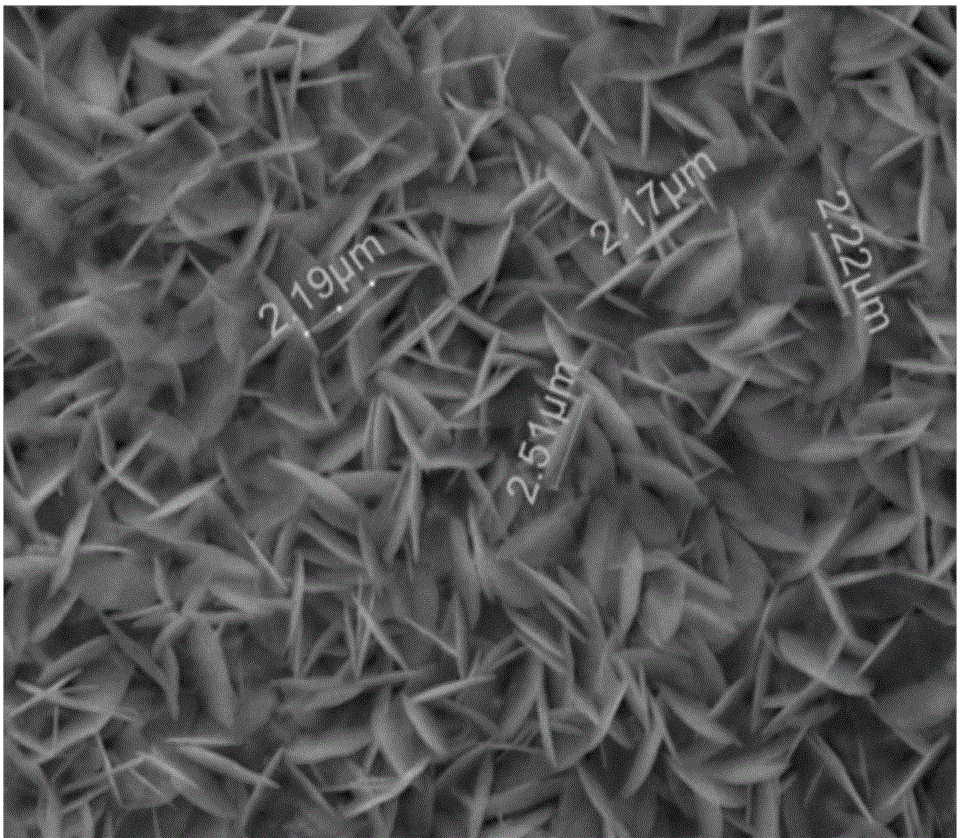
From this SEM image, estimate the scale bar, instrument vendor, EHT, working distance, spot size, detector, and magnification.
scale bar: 5000 nm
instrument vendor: FEI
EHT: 15 kV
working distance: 10.7 mm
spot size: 4.5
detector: ETD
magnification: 10 K X